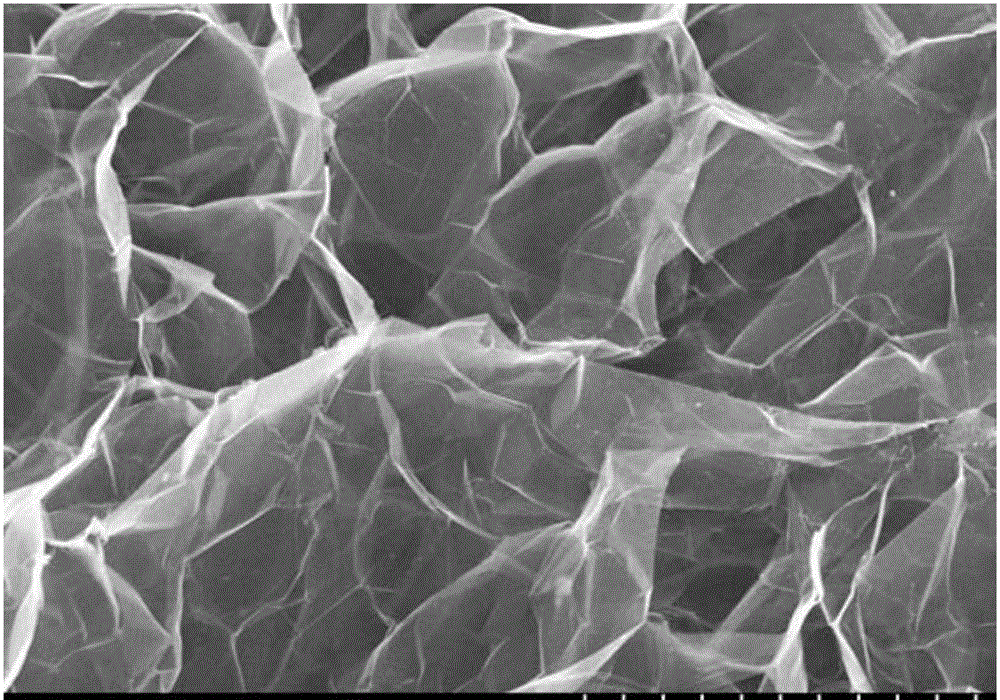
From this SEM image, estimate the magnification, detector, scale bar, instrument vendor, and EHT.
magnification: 10 K X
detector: SE(M)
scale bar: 5000 nm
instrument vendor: Hitachi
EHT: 5 kV